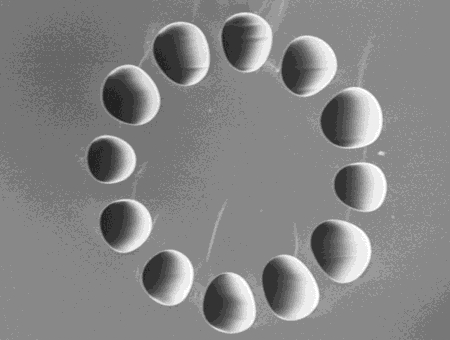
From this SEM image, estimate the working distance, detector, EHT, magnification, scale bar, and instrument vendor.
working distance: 7.8 mm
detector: SE(U)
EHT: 5 kV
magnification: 3 K X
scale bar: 10000 nm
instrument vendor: Hitachi